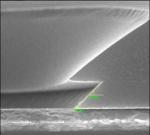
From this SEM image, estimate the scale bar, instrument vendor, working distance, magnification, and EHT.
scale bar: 1000 nm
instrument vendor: Hitachi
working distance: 12.3 mm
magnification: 50 K X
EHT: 15 kV